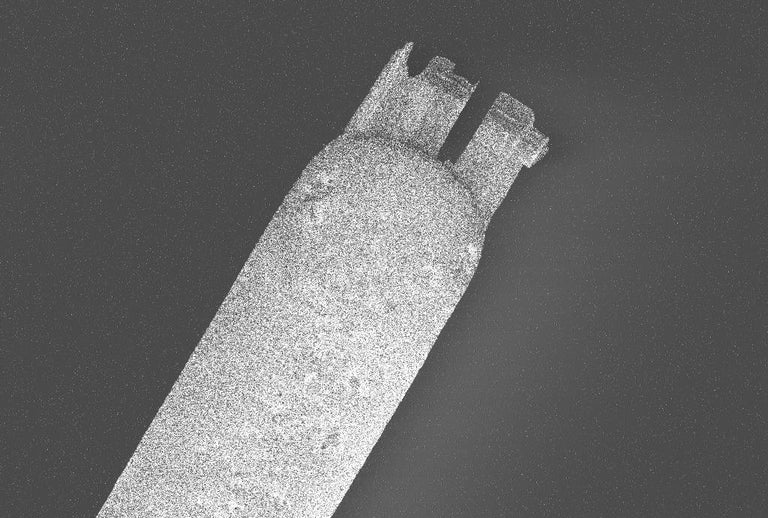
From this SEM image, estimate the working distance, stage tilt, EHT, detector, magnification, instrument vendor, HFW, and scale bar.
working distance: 4.9 mm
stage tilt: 0°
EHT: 2 kV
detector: ESB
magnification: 0.469 K X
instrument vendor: Zeiss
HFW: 243.8 µm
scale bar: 10000 nm